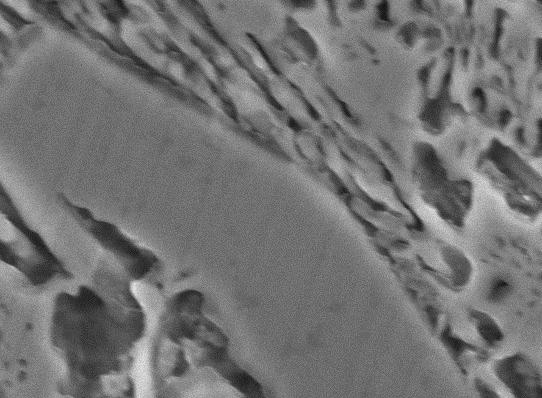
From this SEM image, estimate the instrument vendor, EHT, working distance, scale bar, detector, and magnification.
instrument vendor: JEOL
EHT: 15 kV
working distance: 10.2 mm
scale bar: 1000 nm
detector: SEI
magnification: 10 K X